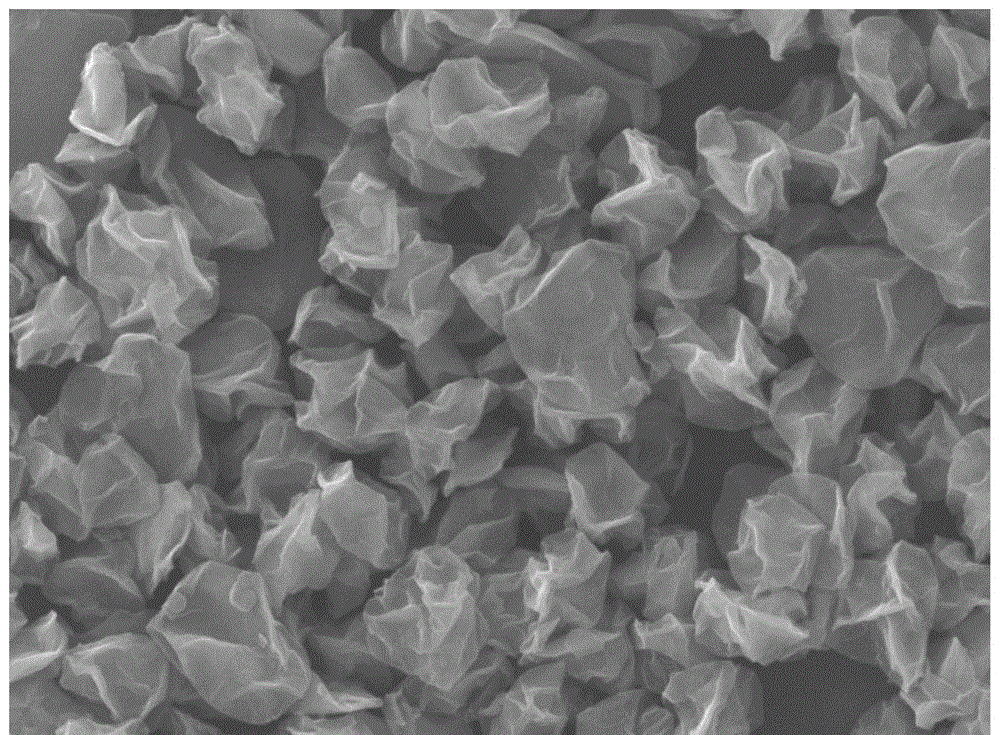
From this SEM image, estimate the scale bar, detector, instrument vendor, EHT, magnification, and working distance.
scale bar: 1000 nm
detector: LED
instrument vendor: JEOL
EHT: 20 kV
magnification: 8 K X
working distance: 9 mm